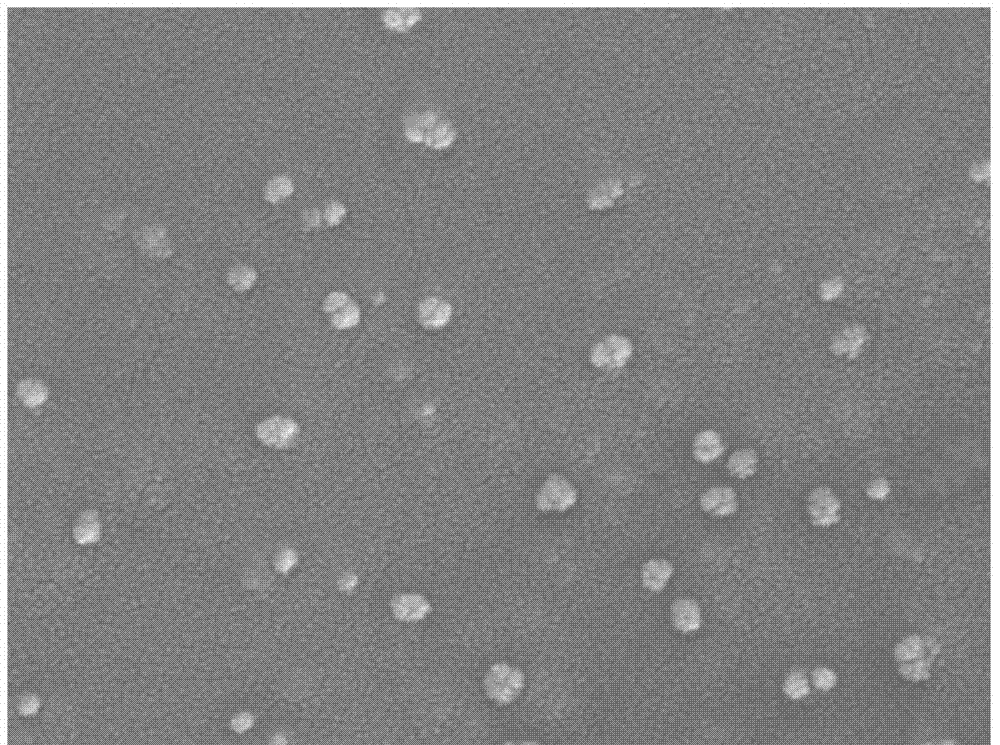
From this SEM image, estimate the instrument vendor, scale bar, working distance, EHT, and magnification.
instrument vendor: JEOL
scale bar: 100 nm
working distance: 6.7 mm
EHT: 5 kV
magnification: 50 K X